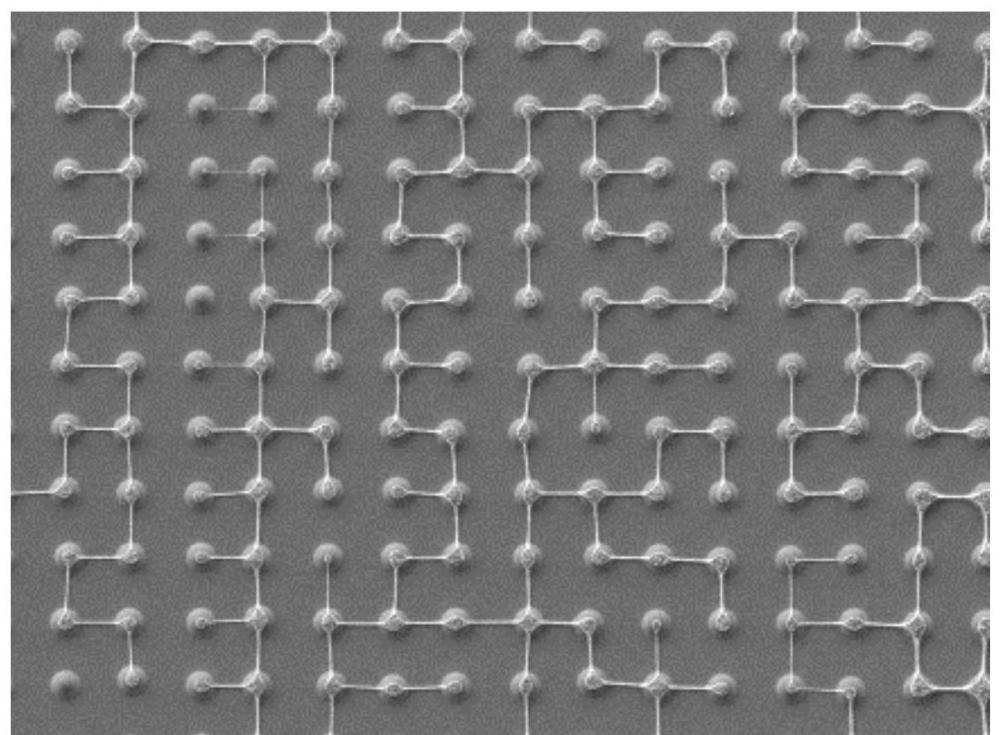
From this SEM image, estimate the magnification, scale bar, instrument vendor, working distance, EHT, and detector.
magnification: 0.2 K X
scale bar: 100000 nm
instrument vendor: JEOL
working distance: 8 mm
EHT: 5 kV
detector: SEI